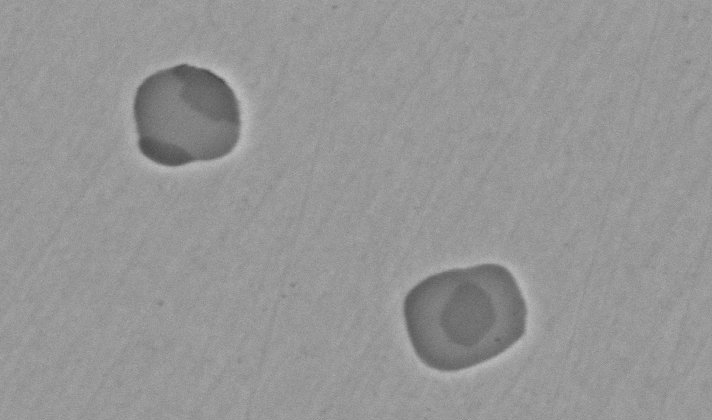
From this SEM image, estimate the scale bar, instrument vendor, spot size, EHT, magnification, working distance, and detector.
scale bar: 5000 nm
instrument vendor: FEI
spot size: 4.5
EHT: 20 kV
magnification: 10 K X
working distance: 10.3 mm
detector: BSE